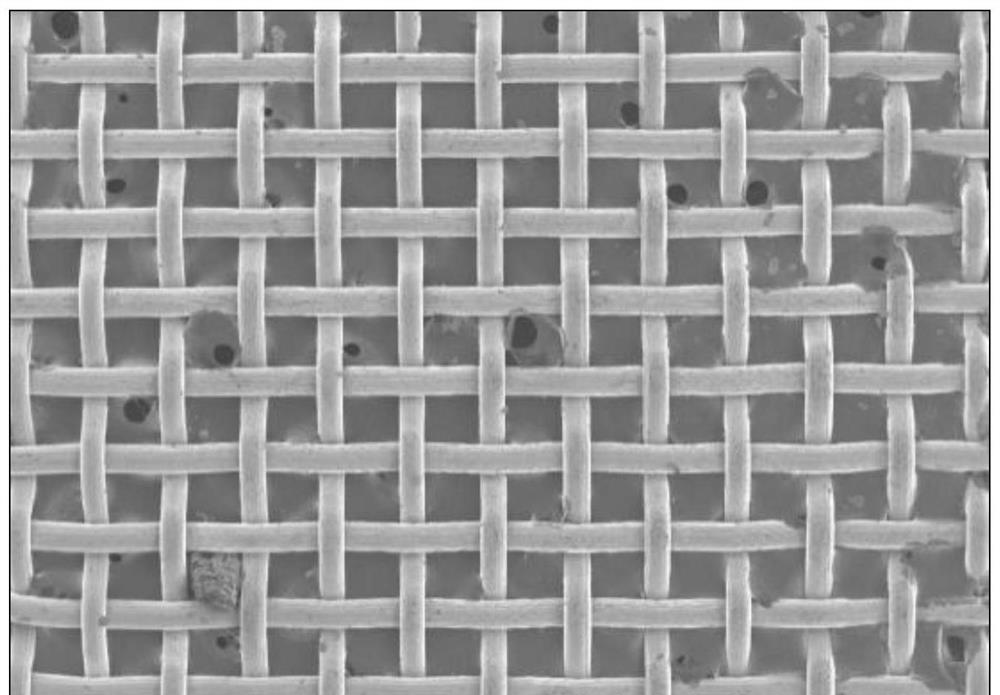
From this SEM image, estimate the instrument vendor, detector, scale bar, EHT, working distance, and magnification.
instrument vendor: Hitachi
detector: SE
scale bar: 1000000 nm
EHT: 3 kV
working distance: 9.5 mm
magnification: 0.05 K X